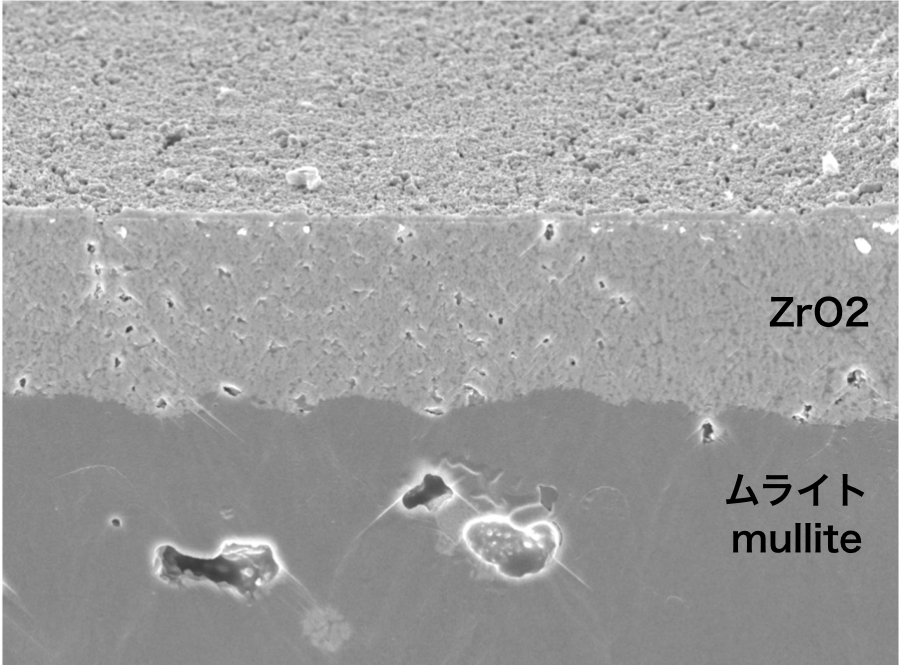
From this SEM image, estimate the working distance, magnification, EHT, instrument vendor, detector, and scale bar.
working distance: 10 mm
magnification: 1 K X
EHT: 15 kV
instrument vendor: JEOL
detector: SEM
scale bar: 10000 nm